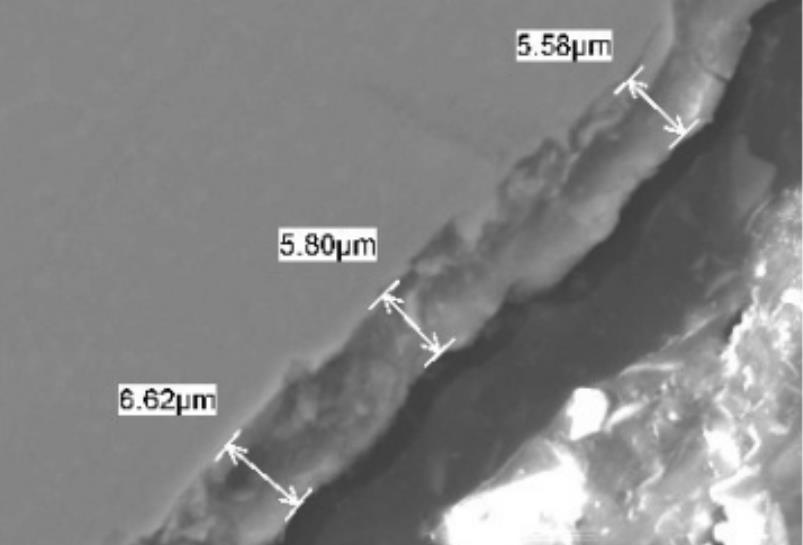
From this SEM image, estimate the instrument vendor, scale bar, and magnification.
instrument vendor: JEOL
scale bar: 10000 nm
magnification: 2 K X